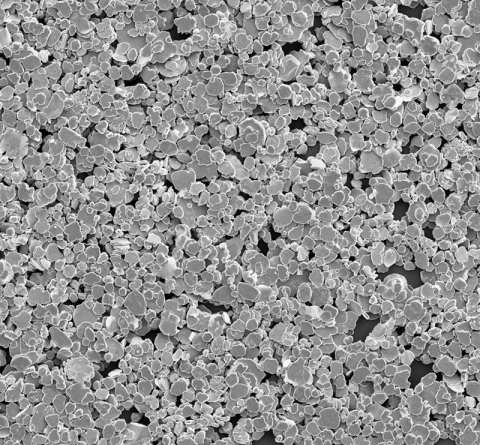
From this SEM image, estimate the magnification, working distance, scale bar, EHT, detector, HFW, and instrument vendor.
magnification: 2 K X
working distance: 7.178 mm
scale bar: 80000 nm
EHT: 10 kV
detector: SED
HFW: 259 µm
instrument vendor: Thermo